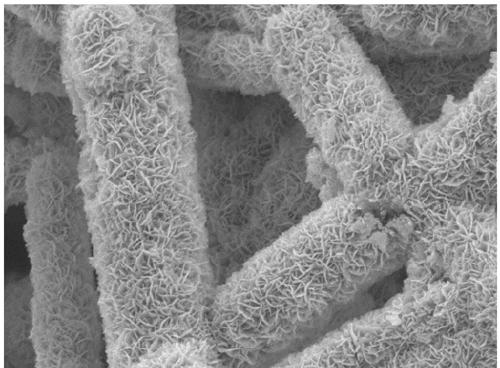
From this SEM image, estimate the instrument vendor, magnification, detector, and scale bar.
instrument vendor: JEOL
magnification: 20 K X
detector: LED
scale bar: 1000 nm